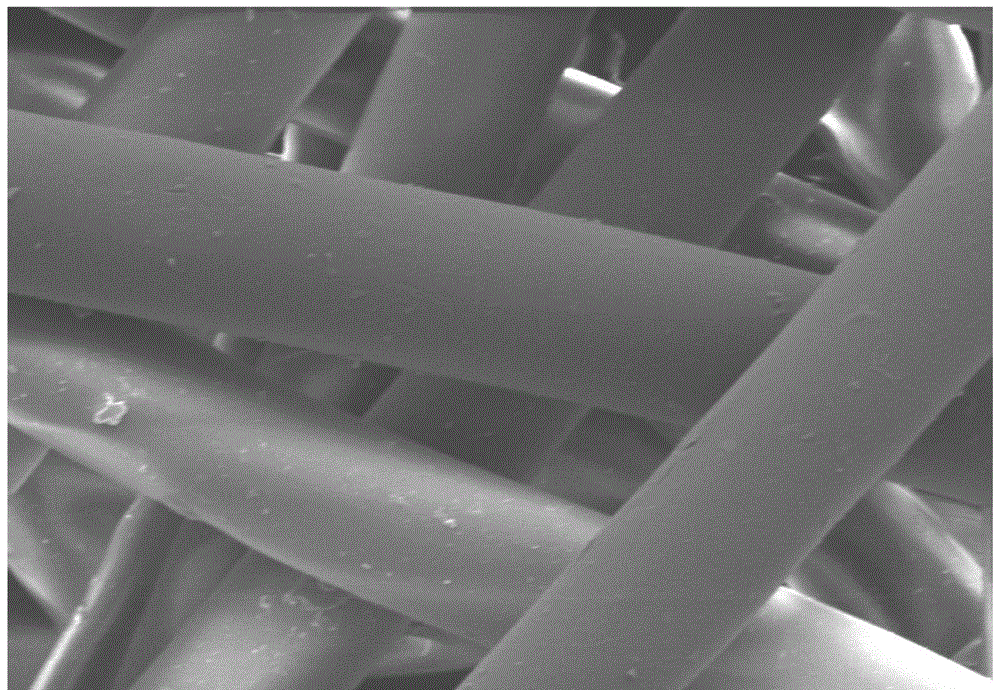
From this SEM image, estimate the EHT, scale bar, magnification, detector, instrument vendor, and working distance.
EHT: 5 kV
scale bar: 50000 nm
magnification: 1 K X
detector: LM(L)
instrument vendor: Hitachi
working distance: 8 mm